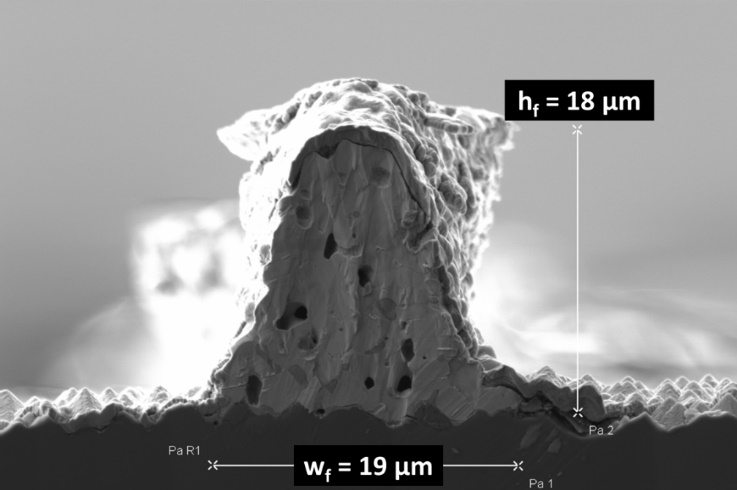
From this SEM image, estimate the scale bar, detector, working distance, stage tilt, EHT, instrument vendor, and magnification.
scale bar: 5000 nm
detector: SE2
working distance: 4.7 mm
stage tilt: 0°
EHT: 5 kV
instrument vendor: Zeiss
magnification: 2.5 K X